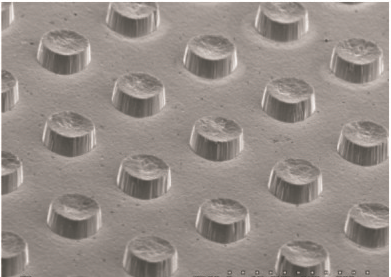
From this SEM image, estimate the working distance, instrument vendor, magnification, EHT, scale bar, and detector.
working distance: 30 mm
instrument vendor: Hitachi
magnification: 0.15 K X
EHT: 15 kV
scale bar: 300000 nm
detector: SE